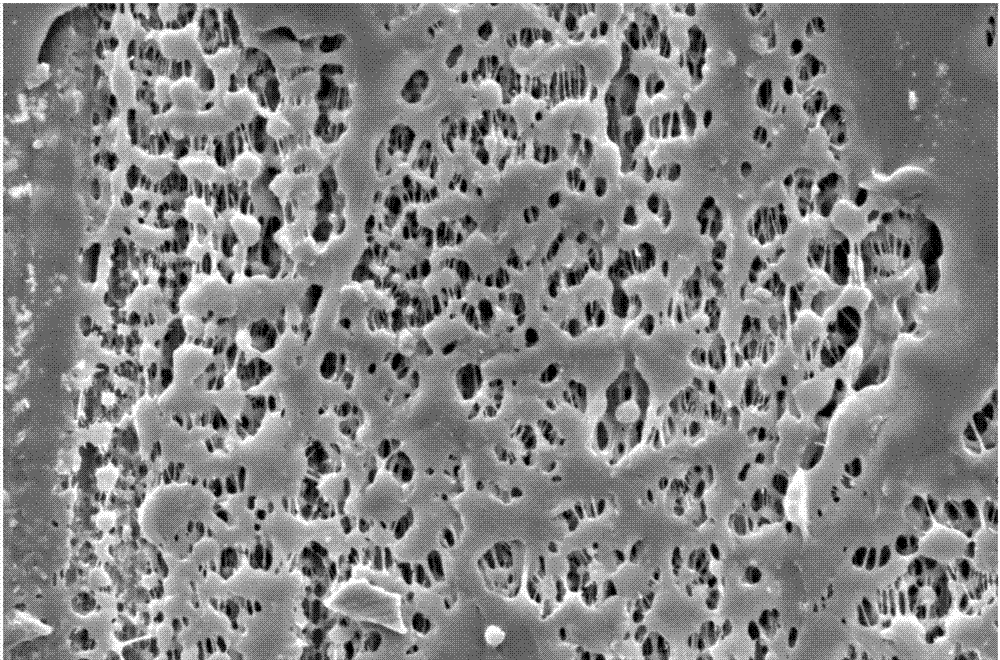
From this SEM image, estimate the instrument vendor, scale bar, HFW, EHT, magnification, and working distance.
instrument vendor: FEI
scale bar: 5000 nm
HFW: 20.7 µm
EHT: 5 kV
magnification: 20 K X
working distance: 10.2 mm